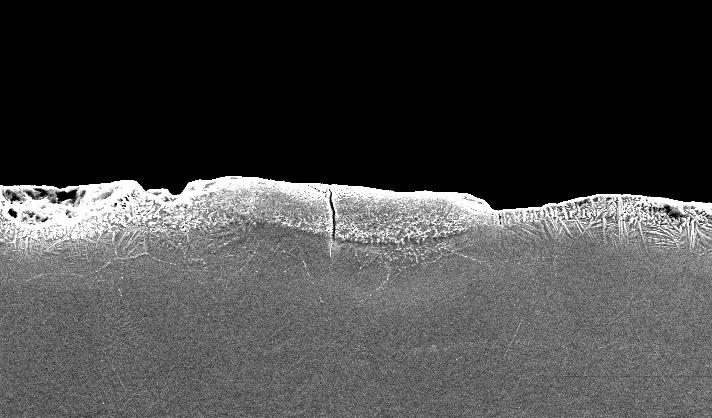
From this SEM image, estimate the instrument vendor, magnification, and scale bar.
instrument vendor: FEI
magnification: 0.25 K X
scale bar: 100000 nm